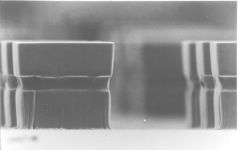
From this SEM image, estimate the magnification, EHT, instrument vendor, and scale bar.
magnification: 0.66 K X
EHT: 10 kV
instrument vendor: JEOL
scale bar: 10000 nm